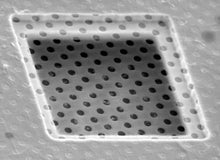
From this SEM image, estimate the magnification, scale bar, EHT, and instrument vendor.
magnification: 2 K X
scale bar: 10000 nm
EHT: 25 kV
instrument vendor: other